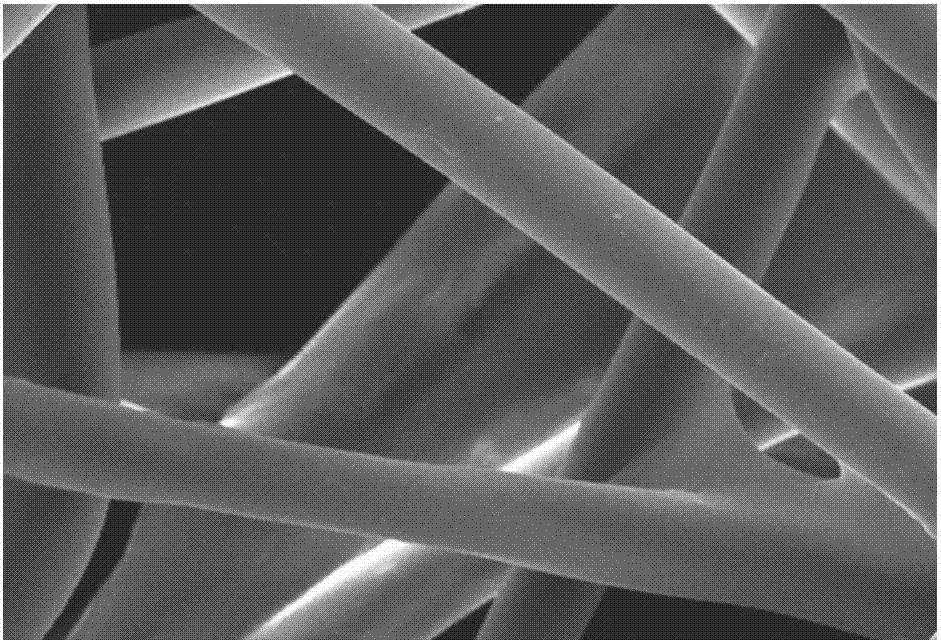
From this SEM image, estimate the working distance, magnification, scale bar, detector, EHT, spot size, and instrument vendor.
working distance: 10 mm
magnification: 1 K X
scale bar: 10000 nm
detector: SEI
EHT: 25 kV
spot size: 30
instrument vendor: JEOL